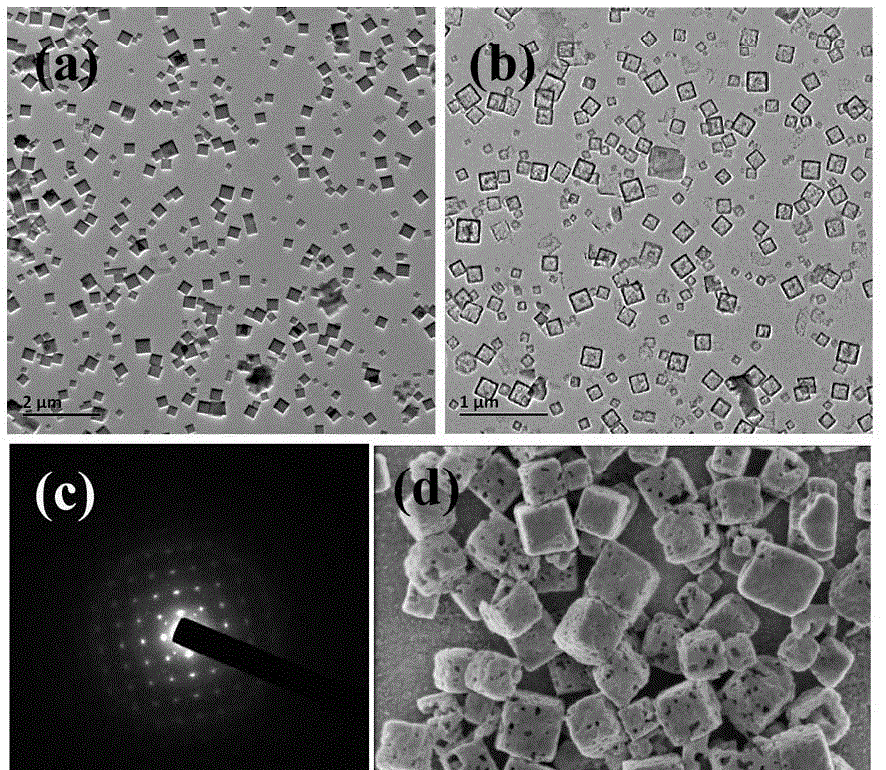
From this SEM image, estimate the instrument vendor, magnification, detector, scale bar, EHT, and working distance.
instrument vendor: FEI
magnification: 200 K X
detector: TLD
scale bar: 500 nm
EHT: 1 kV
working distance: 4.1 mm